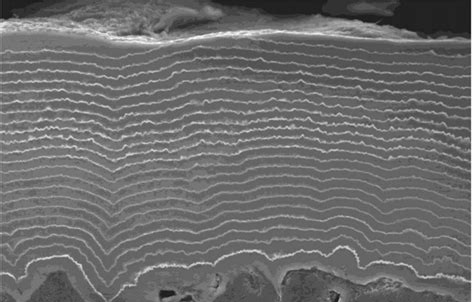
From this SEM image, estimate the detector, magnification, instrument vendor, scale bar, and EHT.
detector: SEI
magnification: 0.85 K X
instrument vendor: JEOL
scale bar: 20000 nm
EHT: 20 kV